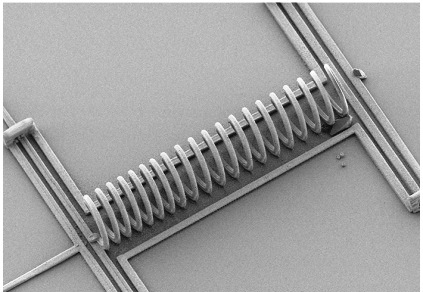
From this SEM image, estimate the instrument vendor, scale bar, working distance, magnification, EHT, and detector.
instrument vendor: Hitachi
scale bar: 100000 nm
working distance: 18 mm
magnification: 0.5 K X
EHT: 1.5 kV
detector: SE(L)